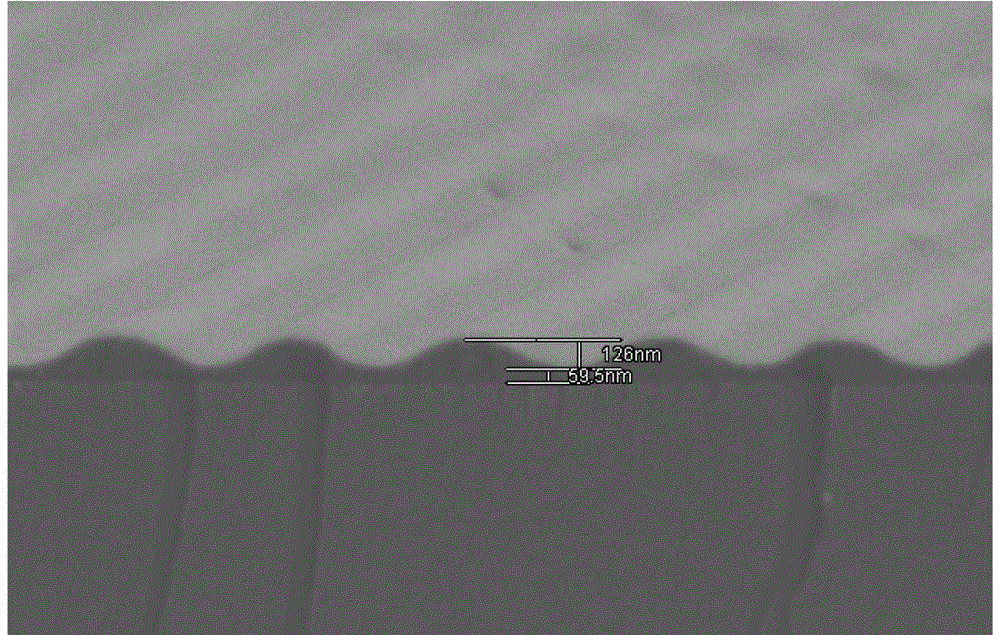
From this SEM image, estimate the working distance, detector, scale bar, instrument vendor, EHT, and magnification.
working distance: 8.1 mm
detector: SE(L)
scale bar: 1000 nm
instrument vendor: Hitachi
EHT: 1.5 kV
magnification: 30 K X